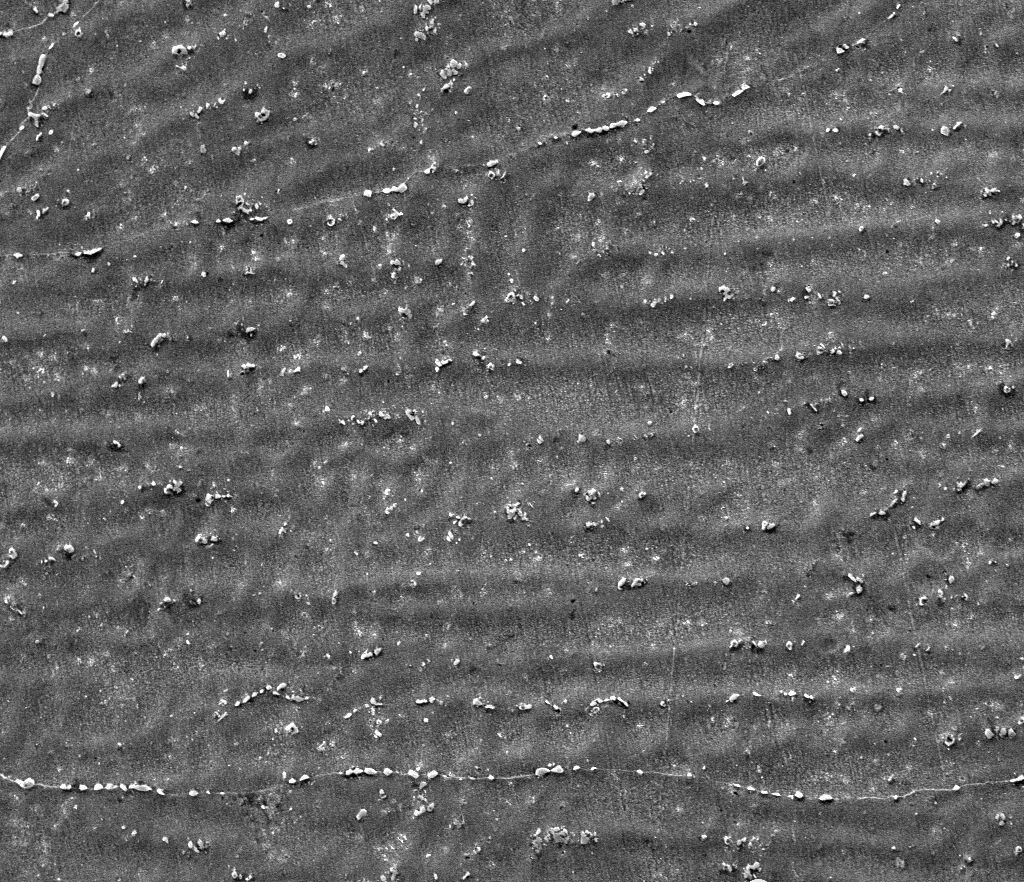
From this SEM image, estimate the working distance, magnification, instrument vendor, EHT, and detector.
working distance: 7.9 mm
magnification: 1 K X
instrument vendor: FEI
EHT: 20 kV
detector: ETD